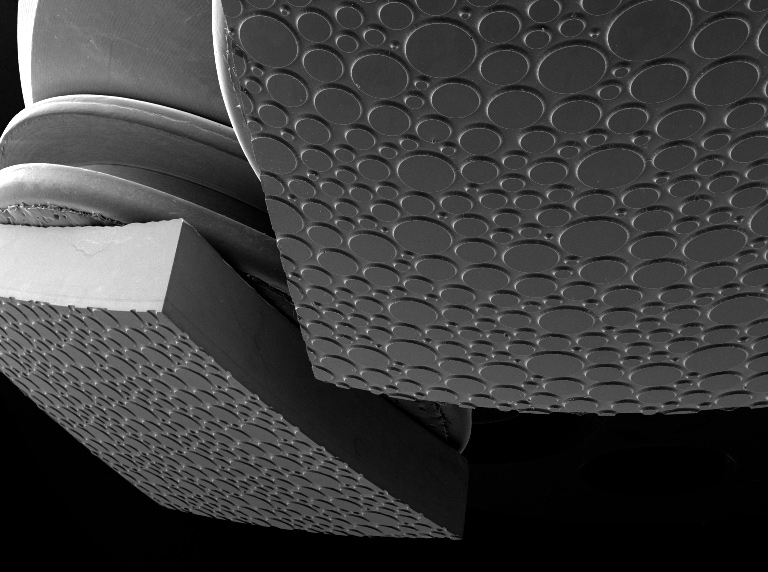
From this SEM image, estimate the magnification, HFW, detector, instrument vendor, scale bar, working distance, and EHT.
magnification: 0.025 K X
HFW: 8670 µm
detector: SE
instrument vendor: Tescan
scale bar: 2000000 nm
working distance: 15.45 mm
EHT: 20 kV